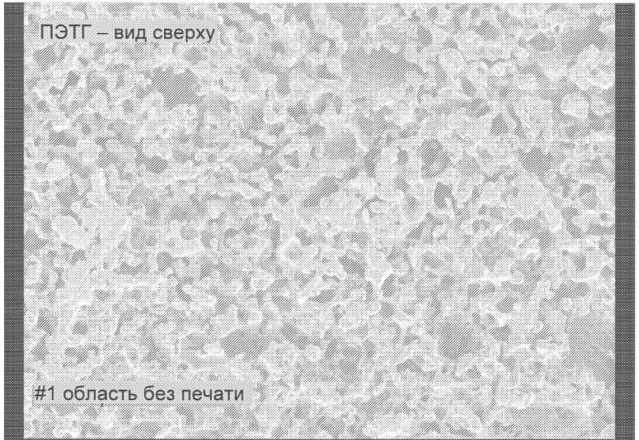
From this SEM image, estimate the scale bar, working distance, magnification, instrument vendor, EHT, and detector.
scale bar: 10000 nm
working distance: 14 mm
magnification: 0.5 K X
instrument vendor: JEOL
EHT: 1 kV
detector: SEI